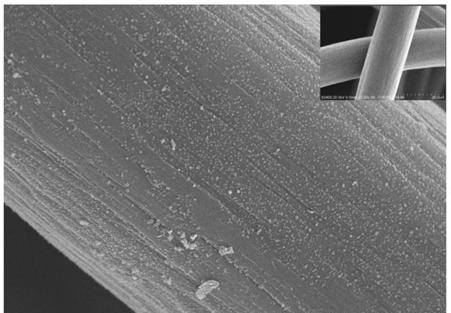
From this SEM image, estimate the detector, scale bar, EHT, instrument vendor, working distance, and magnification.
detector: SE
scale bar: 10000 nm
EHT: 20 kV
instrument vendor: Hitachi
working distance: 7.5 mm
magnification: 5 K X